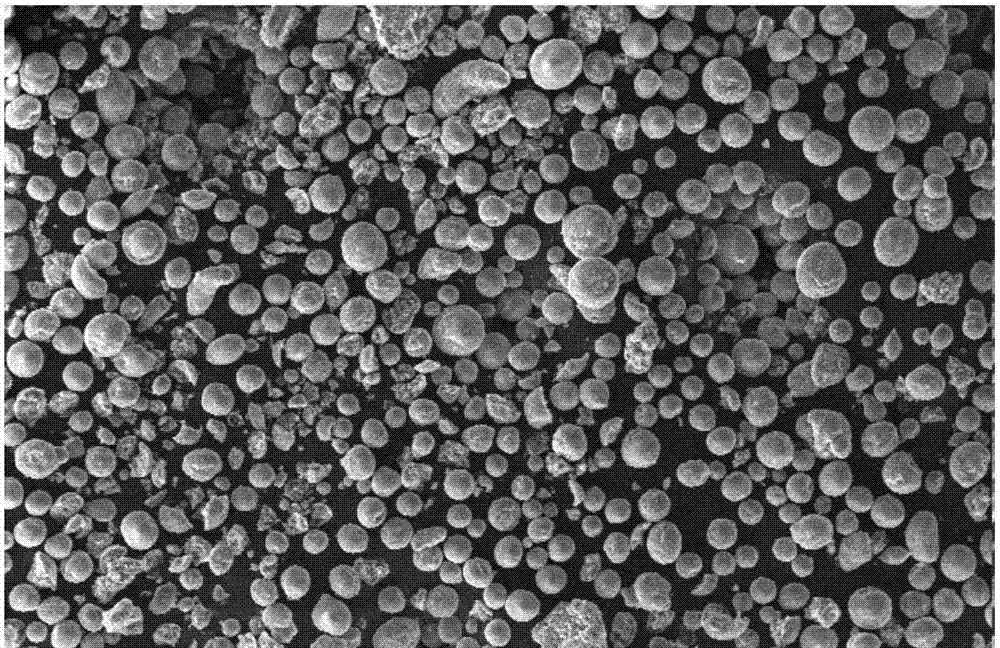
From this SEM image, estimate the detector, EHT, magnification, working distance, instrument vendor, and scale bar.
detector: SE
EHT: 10 kV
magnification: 0.109 K X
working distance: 11 mm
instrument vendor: FEI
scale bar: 200000 nm